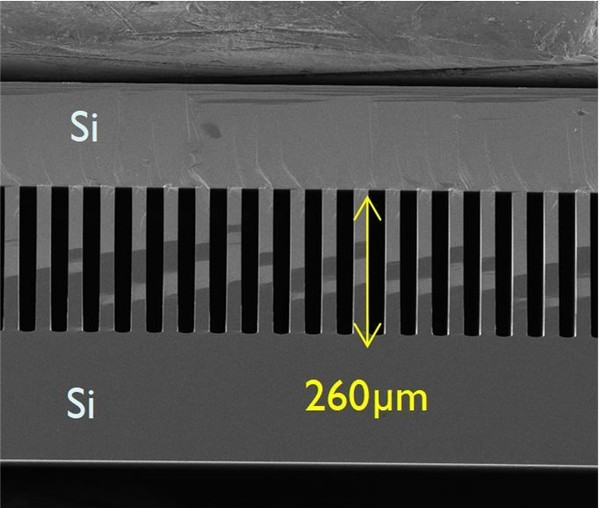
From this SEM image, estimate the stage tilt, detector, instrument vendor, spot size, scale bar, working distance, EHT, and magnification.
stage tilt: -9°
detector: ETD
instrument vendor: FEI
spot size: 3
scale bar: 400000 nm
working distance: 6.5 mm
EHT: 5 kV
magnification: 0.225 K X